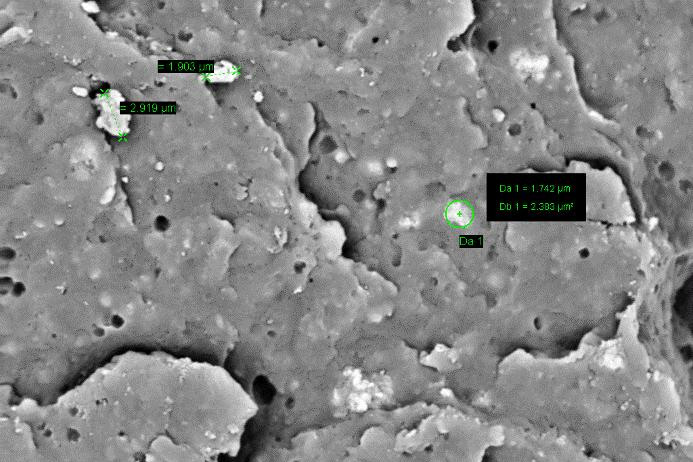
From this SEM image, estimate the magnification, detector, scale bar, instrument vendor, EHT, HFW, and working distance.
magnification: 7 K X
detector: C2 BSD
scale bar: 2000 nm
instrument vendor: Zeiss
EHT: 10 kV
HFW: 42.47 µm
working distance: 8 mm